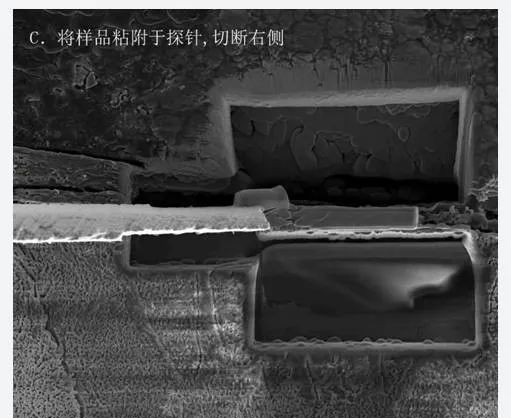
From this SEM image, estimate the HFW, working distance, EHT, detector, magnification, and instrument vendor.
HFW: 36.6 µm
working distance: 4 mm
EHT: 5 kV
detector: ETD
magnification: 7 K X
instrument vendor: FEI